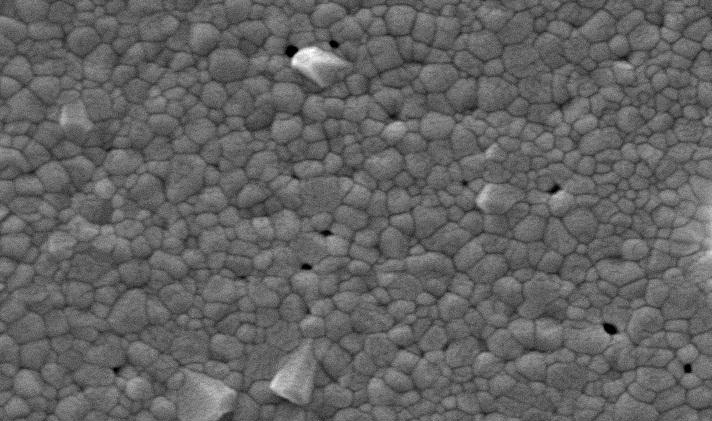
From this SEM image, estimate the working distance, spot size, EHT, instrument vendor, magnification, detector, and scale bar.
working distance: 8 mm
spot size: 4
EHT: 20 kV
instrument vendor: FEI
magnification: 10 K X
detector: SE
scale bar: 2000 nm